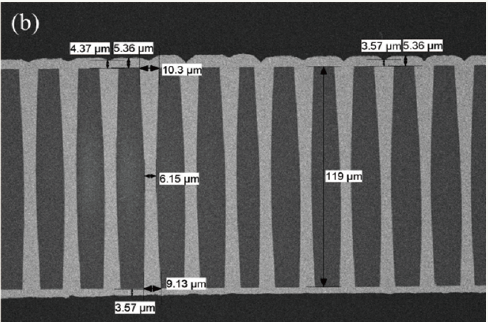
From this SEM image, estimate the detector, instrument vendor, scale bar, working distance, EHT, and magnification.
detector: HA3(T)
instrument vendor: Hitachi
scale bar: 100000 nm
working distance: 8.1 mm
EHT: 3 kV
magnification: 0.5 K X